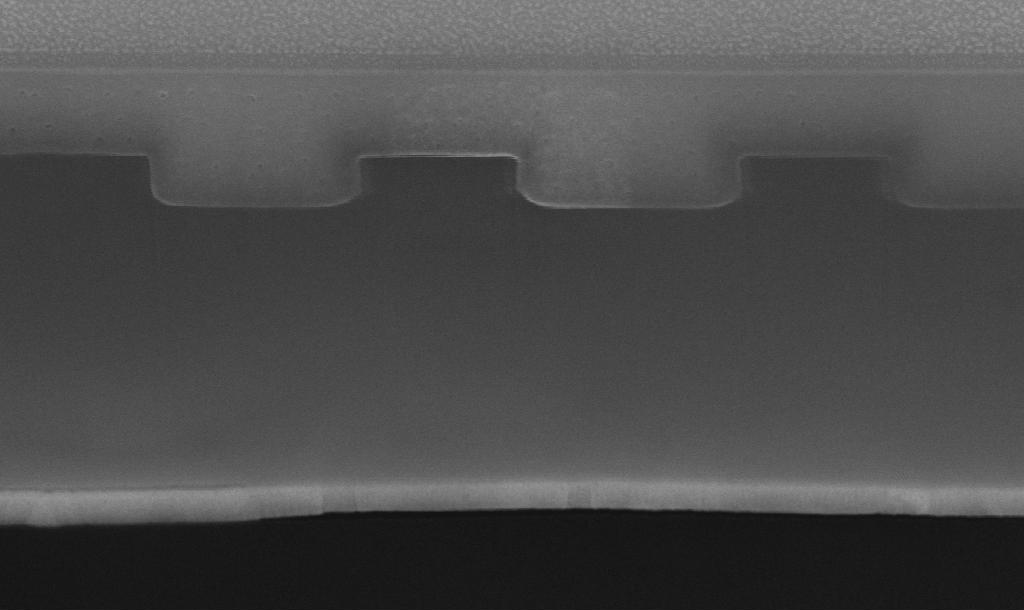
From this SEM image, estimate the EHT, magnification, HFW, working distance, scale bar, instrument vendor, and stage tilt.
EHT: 18 kV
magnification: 150 K X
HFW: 1.71 µm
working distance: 5.1 mm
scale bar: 500 nm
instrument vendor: FEI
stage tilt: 52°